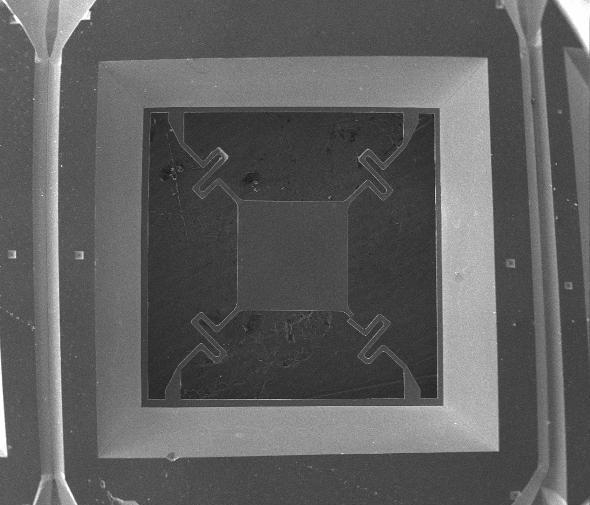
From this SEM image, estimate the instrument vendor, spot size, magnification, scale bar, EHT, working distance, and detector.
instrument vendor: FEI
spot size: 3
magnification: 0.025 K X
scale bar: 2000000 nm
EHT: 20 kV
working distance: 13.8 mm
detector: ETD